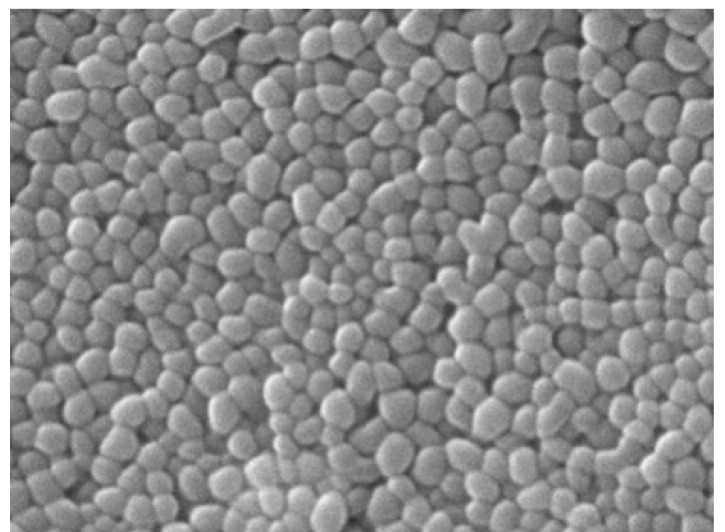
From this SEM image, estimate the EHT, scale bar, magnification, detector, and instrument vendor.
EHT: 7 kV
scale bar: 500 nm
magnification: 40 K X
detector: SED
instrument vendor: JEOL